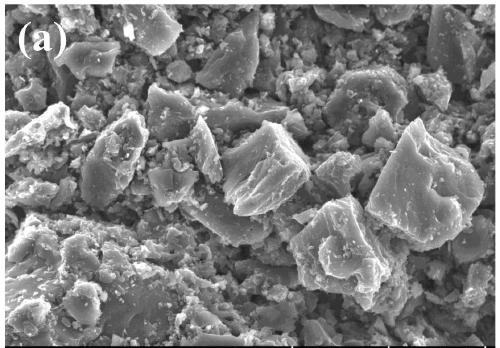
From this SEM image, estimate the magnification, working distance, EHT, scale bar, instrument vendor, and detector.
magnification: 1 K X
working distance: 9 mm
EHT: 15 kV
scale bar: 50000 nm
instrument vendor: Hitachi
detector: SE(UL)